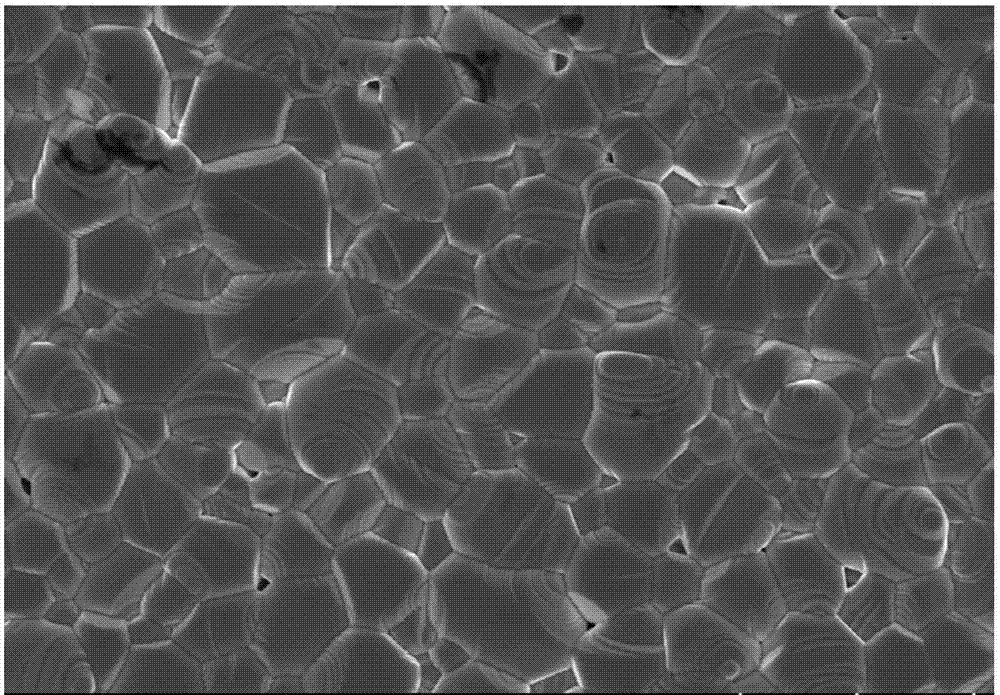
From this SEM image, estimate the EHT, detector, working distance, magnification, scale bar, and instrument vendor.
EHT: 10 kV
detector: SE(U)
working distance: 10.5 mm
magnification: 3 K X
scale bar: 10000 nm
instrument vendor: Hitachi